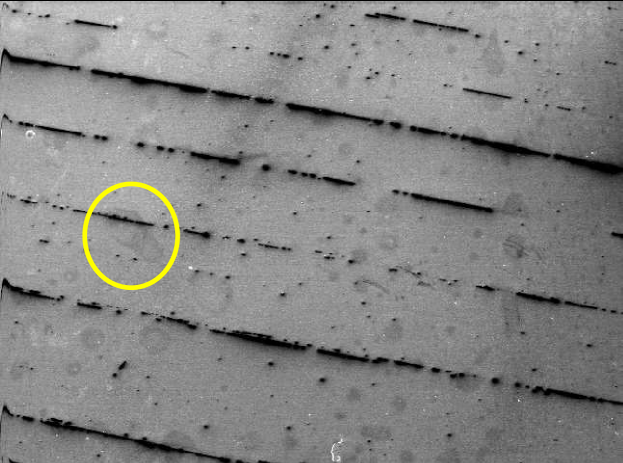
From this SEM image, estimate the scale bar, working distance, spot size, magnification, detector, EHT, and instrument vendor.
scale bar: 500000 nm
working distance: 10.1 mm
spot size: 5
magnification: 0.049 K X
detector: SE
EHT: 20 kV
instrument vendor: FEI